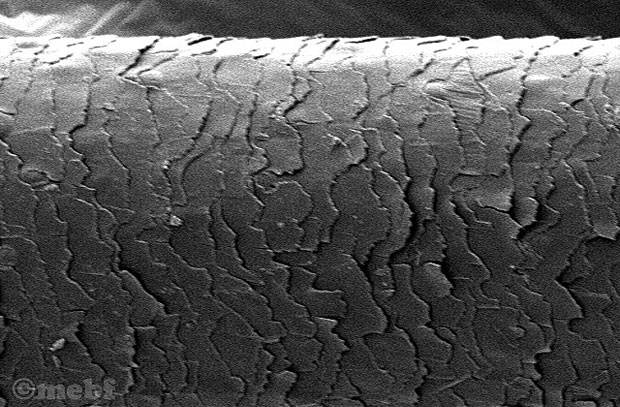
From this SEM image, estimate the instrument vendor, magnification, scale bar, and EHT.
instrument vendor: other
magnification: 0.94 K X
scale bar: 10000 nm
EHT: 5 kV